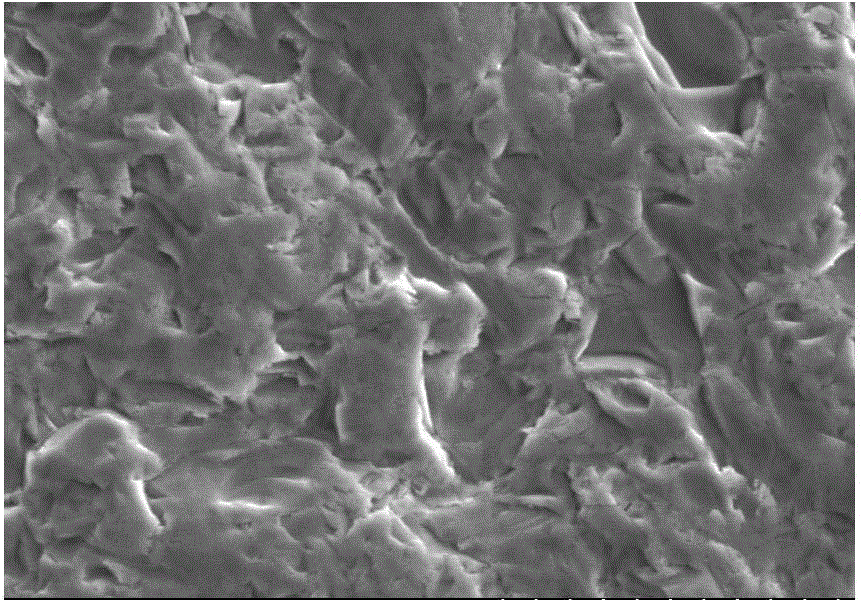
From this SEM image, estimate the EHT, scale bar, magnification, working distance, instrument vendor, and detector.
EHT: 6 kV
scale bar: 10000 nm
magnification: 5 K X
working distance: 10.8 mm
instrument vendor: Hitachi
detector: SE